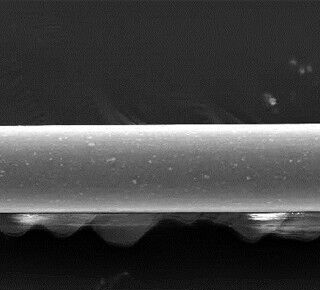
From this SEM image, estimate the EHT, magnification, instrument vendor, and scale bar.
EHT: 20 kV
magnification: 0.5 K X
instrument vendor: JEOL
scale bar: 50000 nm